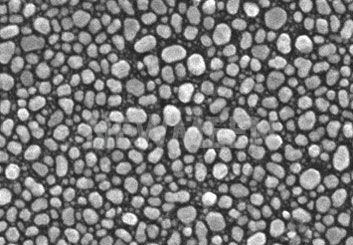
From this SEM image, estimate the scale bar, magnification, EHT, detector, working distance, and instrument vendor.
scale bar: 500 nm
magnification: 60 K X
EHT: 20 kV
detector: SE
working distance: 5 mm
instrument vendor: Hitachi